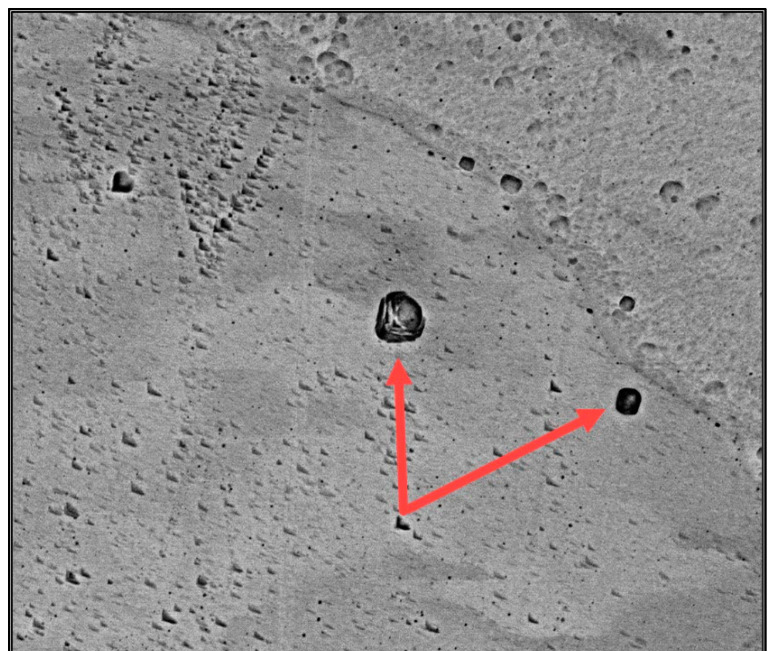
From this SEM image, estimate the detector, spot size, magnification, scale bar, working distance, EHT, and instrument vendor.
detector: BSED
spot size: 6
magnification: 5 K X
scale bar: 10000 nm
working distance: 10 mm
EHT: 20 kV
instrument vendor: FEI